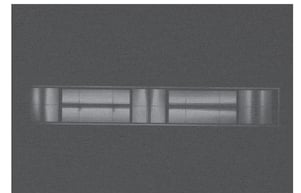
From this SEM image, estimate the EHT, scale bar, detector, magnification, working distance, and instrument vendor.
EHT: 2 kV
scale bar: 50000 nm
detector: SE(M)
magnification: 0.6 K X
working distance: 2.7 mm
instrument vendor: Hitachi